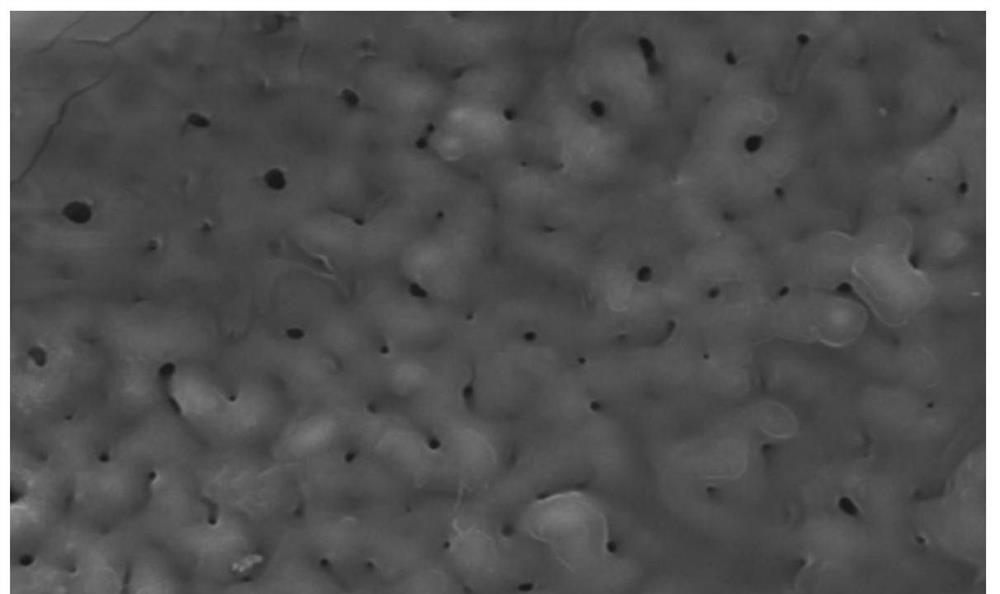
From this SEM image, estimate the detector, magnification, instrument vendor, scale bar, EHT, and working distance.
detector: LED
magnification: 2 K X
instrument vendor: JEOL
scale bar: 10000 nm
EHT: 15 kV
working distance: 10.7 mm